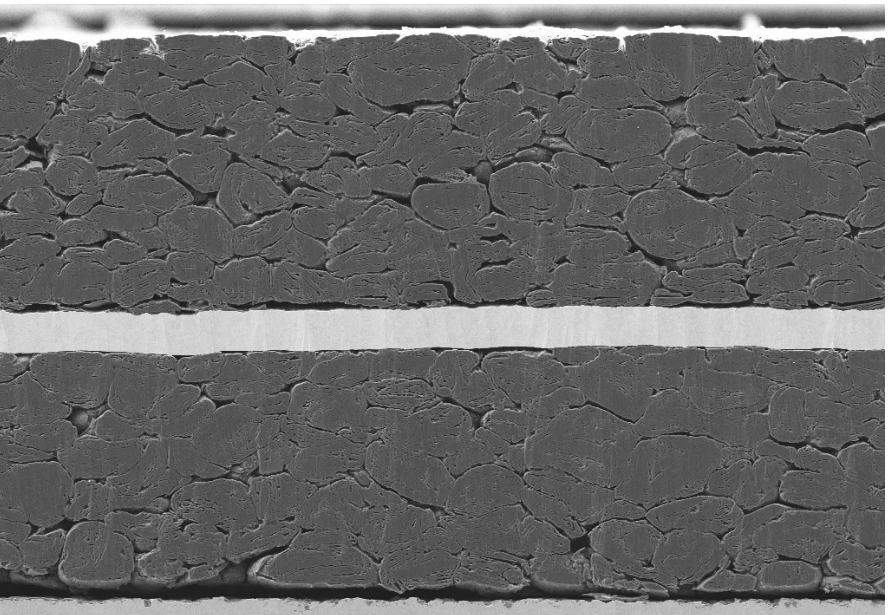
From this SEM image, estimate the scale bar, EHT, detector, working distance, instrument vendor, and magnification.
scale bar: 10000 nm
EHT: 5 kV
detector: SE2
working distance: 5.1 mm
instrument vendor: Zeiss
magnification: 0.55 K X